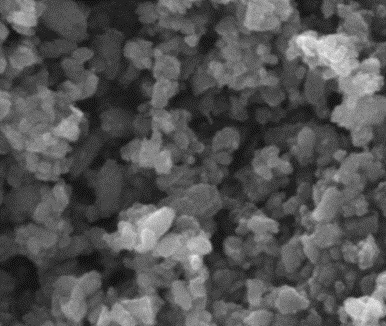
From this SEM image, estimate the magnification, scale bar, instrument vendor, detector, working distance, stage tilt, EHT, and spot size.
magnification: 100 K X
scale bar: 500 nm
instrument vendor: FEI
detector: ETD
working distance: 10.6 mm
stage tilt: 0°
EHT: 20 kV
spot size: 1.5